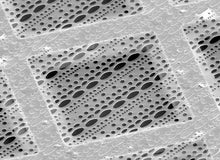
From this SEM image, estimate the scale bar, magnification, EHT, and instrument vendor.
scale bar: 30000 nm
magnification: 1 K X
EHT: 25 kV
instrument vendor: other